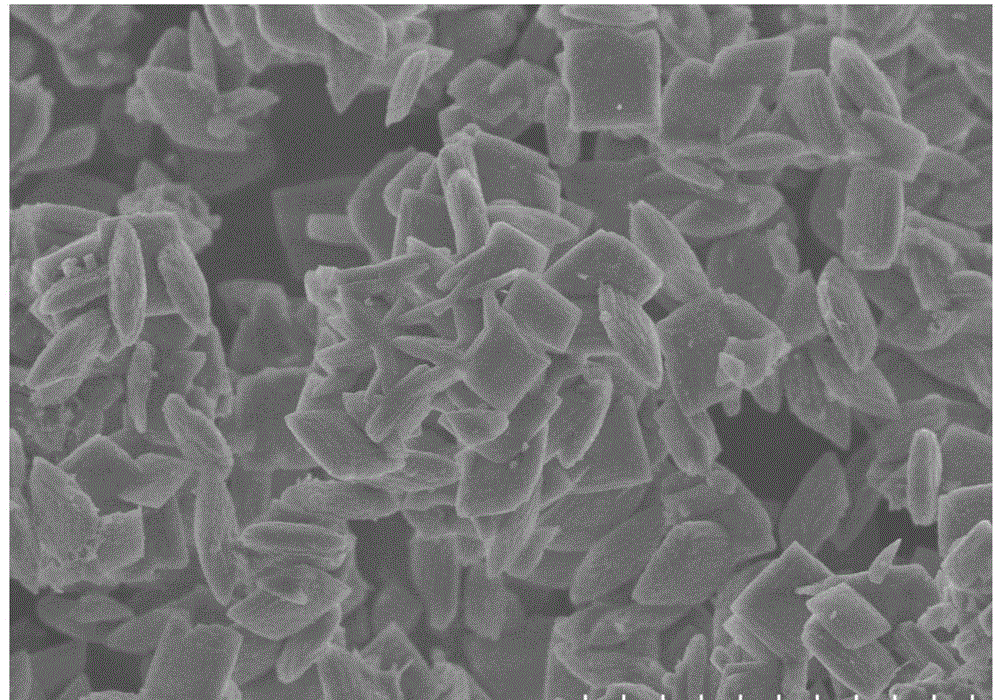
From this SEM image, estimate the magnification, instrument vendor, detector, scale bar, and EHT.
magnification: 5 K X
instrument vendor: Hitachi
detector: SE(M)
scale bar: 10000 nm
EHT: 5 kV